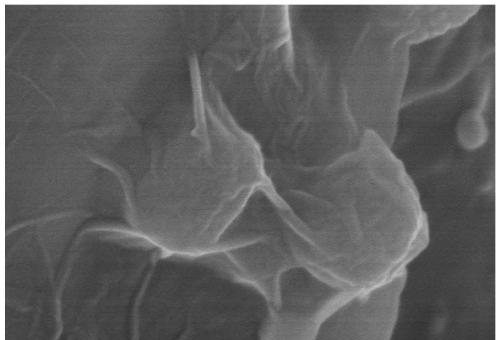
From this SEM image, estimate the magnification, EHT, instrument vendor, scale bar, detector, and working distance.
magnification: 130 K X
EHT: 5 kV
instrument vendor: Hitachi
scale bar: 400 nm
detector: SE(UL)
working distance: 8.6 mm